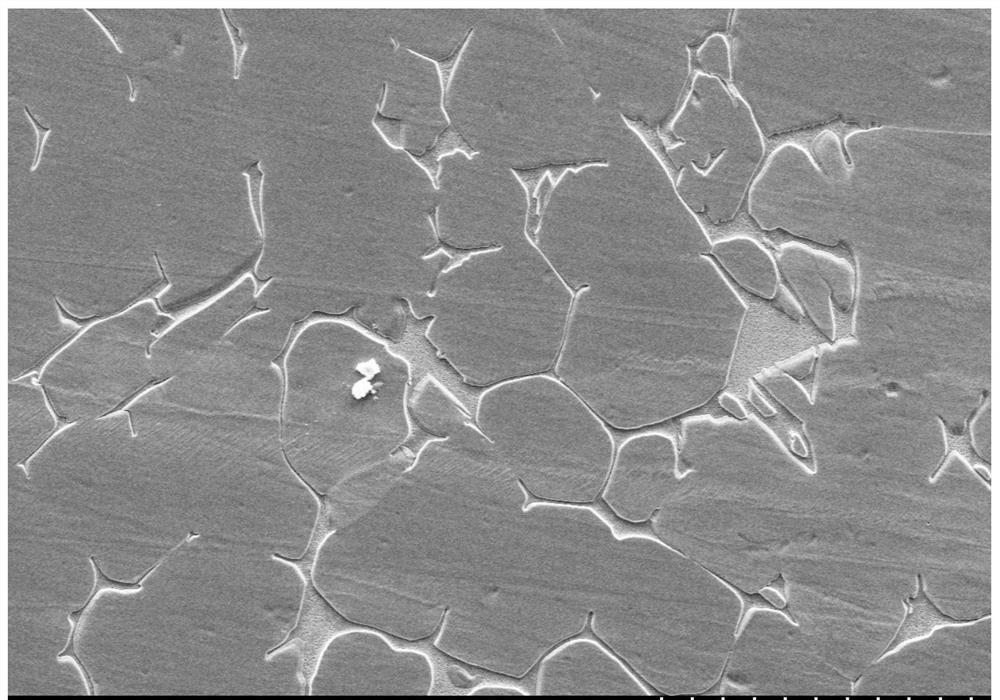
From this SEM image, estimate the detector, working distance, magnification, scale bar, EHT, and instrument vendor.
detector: SE(M)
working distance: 39.2 mm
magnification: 0.2 K X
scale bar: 200000 nm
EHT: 20 kV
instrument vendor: Hitachi